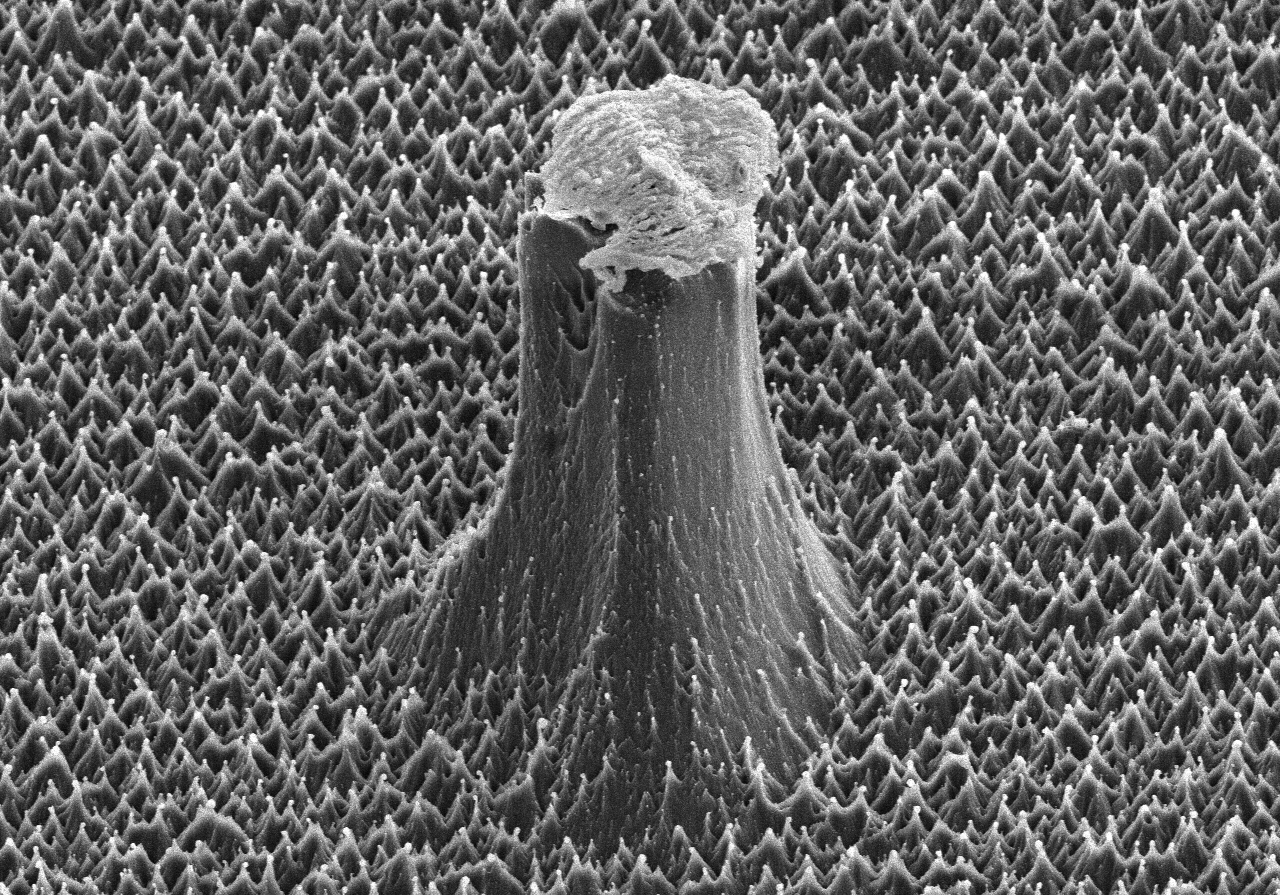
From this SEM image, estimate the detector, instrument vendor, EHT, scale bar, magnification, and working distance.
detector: SE(M)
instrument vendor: Hitachi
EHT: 6 kV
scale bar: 20000 nm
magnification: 2 K X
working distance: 12.8 mm